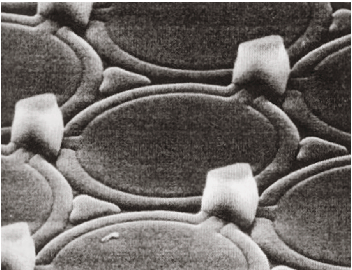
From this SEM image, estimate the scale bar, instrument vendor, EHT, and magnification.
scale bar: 6670 nm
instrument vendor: JEOL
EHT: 15 kV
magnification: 4.5 K X